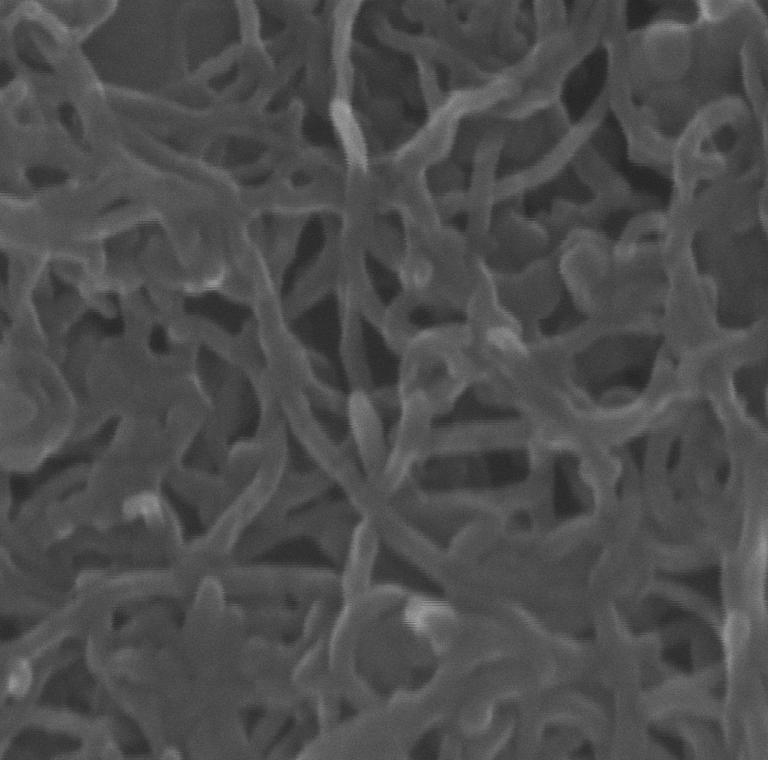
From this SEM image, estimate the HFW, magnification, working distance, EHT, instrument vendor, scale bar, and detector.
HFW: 0.693 µm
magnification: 312.75 K X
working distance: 3.464 mm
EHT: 15 kV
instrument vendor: Tescan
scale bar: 200 nm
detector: InBeam